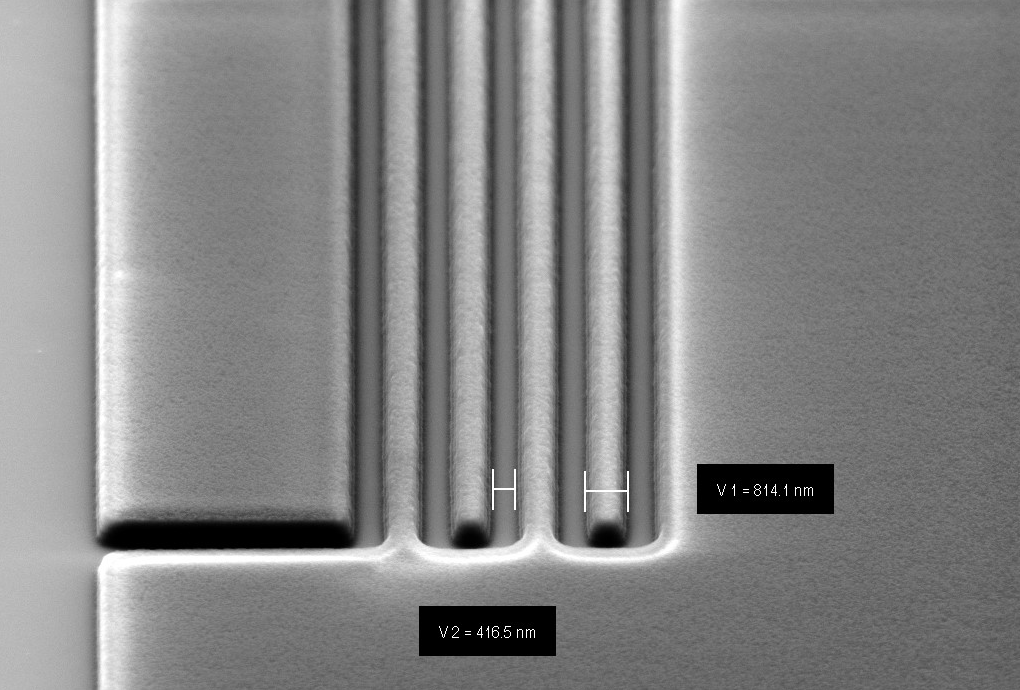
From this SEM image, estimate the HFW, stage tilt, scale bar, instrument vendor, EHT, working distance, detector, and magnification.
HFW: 19.39 µm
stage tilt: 36°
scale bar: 1000 nm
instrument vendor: Zeiss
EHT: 5 kV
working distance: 5.8 mm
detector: SE2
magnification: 51.59 K X